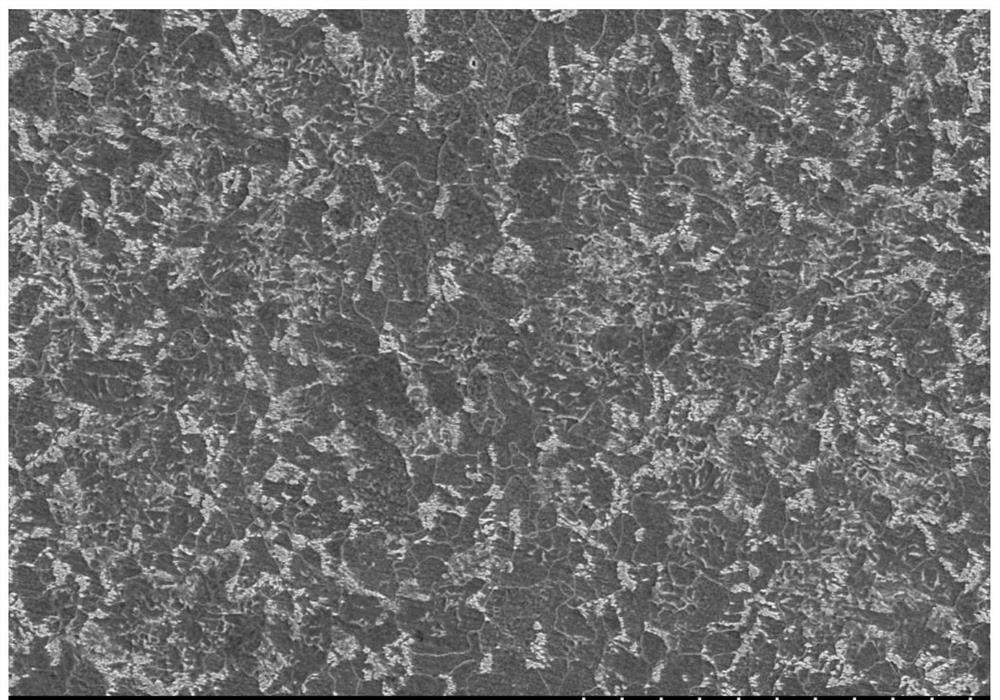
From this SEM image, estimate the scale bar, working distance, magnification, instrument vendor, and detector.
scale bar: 50000 nm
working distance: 9.5 mm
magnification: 1 K X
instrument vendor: Hitachi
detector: SE